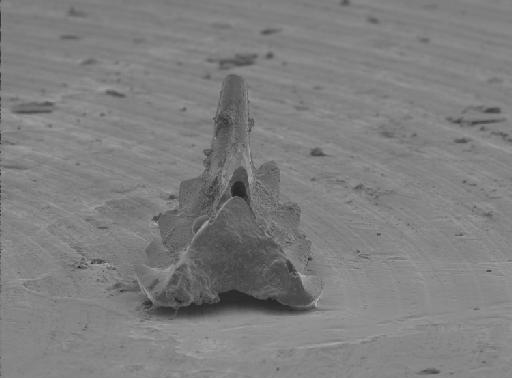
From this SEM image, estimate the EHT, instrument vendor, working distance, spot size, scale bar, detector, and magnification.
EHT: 5 kV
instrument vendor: FEI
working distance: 10.1 mm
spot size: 3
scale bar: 100000 nm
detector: SE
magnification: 0.2 K X